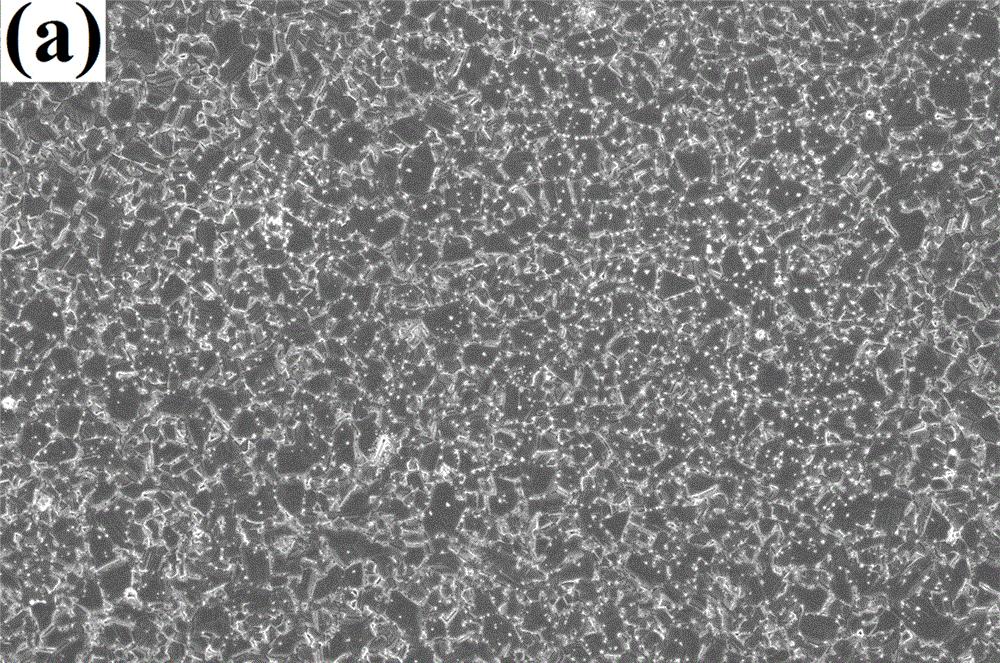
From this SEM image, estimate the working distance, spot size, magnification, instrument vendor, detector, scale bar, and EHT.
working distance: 4.8 mm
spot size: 3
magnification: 10 K X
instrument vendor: FEI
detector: TLD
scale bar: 10000 nm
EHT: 5 kV